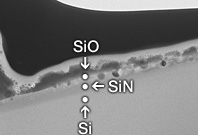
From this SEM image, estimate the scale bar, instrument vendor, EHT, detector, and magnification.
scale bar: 300 nm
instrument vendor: Hitachi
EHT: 200 kV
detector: TE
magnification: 100 K X